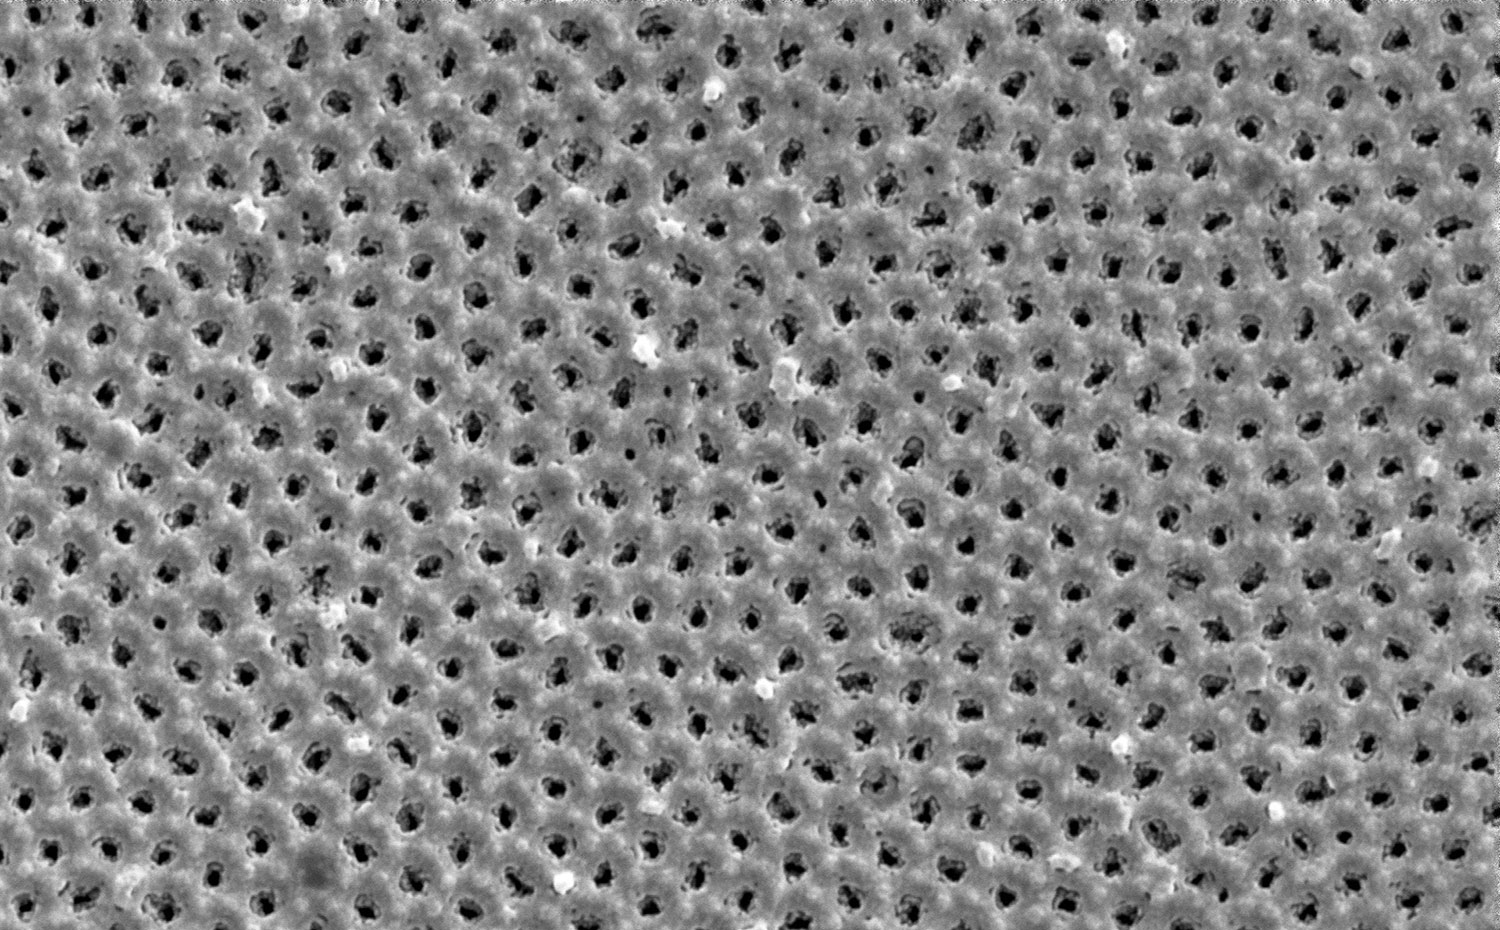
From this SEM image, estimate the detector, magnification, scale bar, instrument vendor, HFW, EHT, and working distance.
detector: SED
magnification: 200 K X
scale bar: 800 nm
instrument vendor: Thermo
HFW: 2.59 µm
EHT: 20 kV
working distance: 5.838 mm